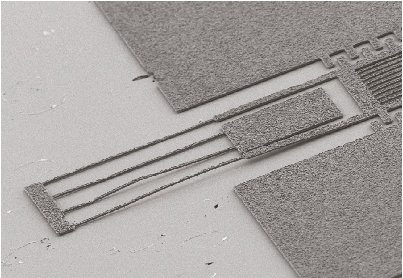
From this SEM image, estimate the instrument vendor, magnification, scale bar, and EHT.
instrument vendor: Hitachi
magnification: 0.5 K X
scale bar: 60000 nm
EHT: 5 kV